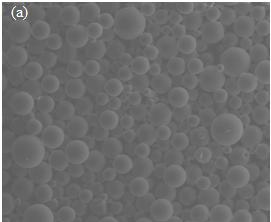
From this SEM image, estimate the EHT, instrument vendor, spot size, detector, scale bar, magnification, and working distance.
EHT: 25 kV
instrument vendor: FEI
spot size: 5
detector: LFD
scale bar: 500000 nm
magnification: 0.1 K X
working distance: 13.1 mm